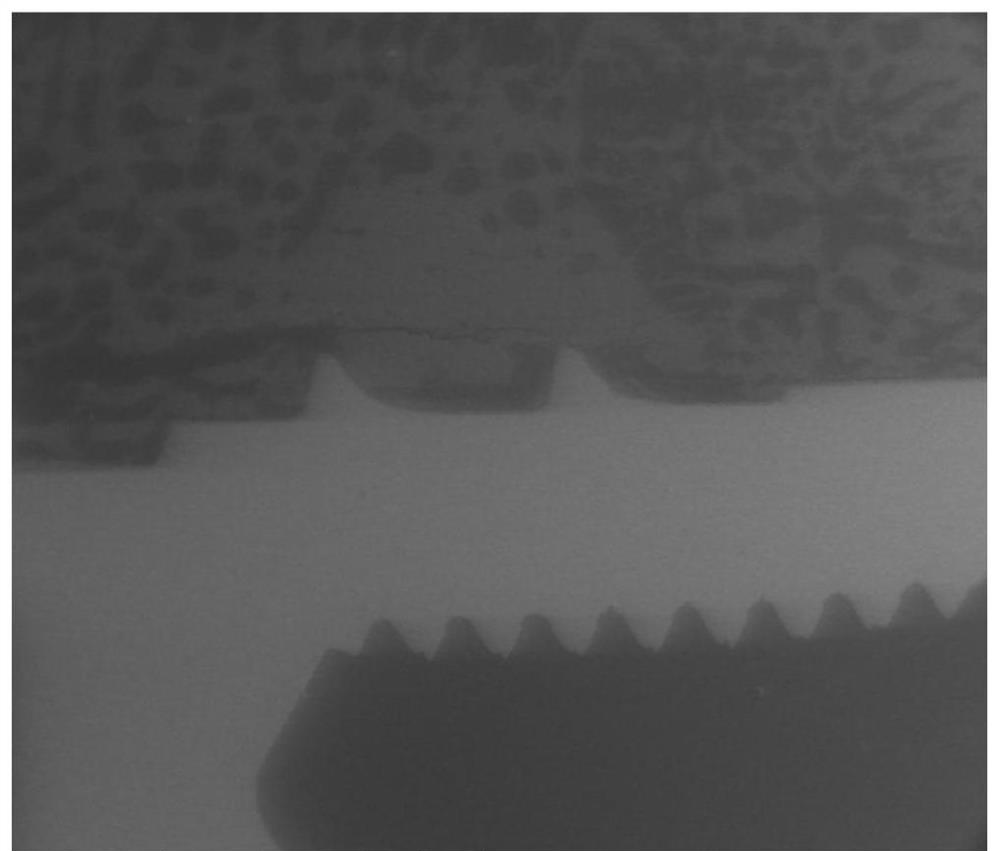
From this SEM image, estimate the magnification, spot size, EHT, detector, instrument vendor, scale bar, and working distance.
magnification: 0.05 K X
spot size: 4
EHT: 20 kV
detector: LFD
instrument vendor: FEI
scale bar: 2000000 nm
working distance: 9.4 mm